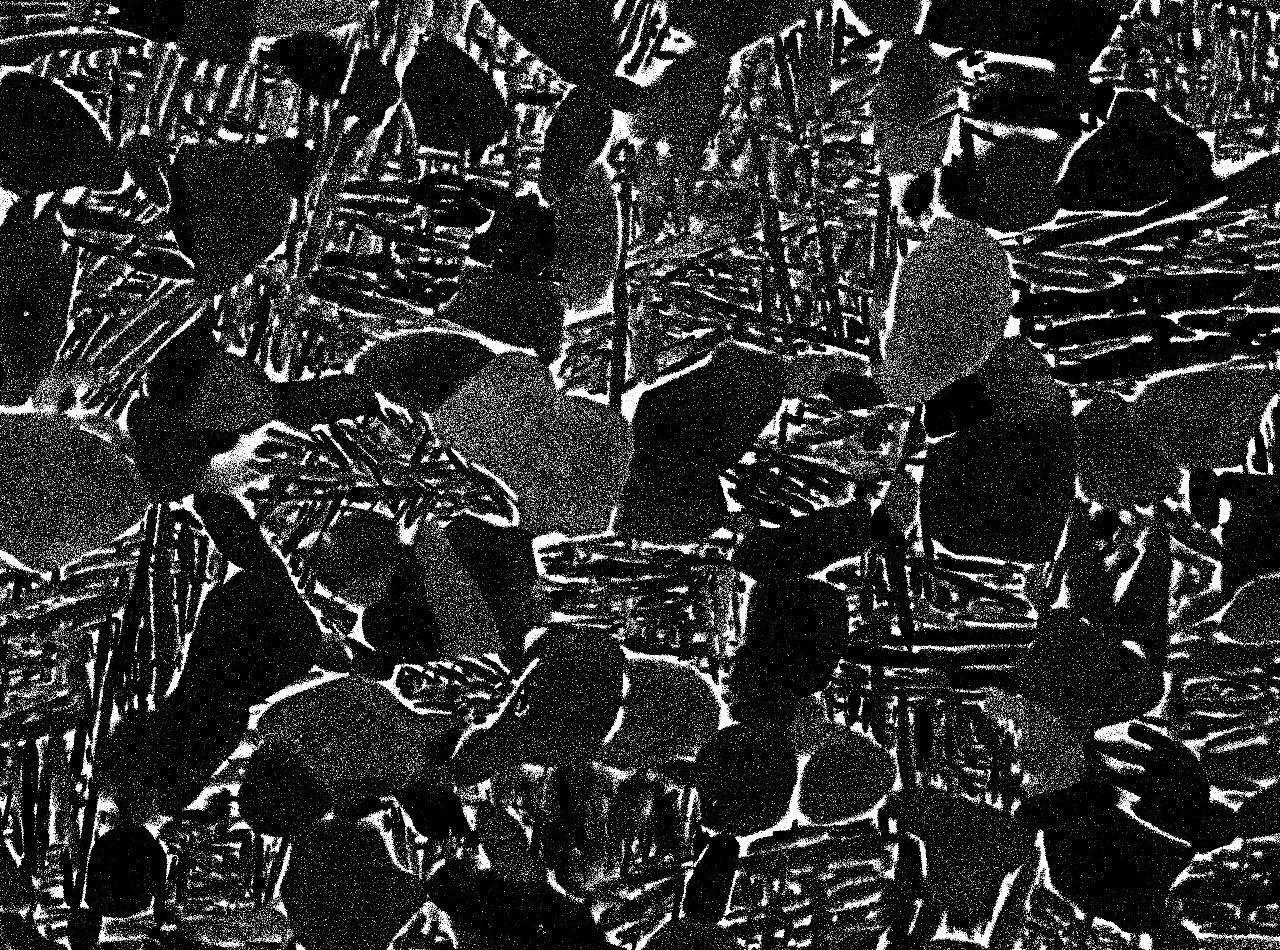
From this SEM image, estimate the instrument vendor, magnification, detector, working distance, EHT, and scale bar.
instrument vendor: JEOL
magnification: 2 K X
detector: COMPO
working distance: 10 mm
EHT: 15 kV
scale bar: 10000 nm